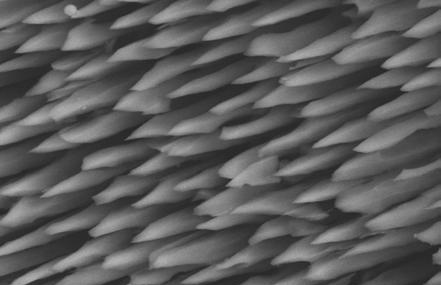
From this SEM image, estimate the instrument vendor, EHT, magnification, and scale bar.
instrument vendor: other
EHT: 20 kV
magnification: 1.5 K X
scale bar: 6660 nm